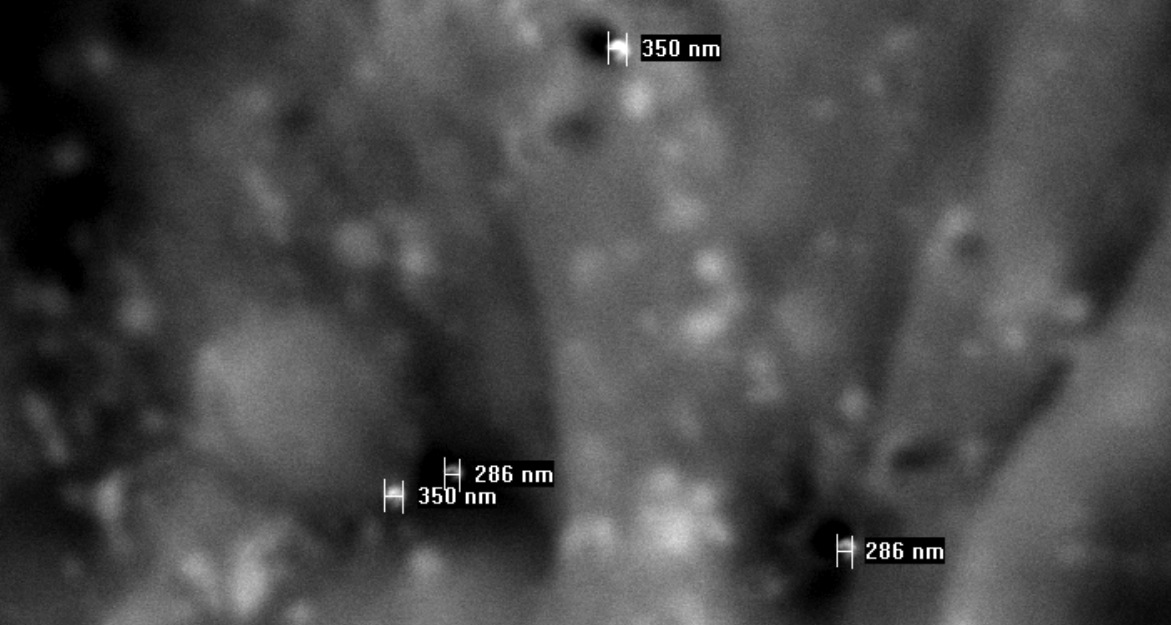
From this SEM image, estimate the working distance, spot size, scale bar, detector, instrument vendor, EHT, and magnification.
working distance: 10.5 mm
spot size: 5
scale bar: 5000 nm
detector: BSE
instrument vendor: FEI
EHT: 20 kV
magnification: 5.218 K X